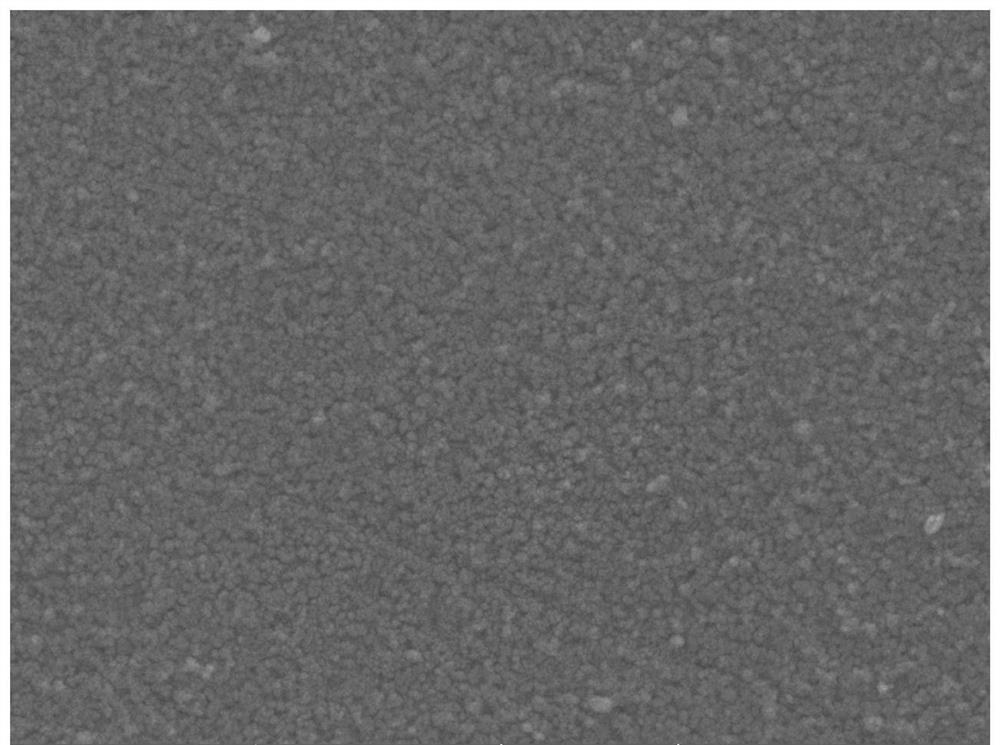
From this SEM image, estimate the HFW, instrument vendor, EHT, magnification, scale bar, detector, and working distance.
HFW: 5.54 µm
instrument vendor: Tescan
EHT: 5 kV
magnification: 50 K X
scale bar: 1000 nm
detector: SE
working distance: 6.47 mm